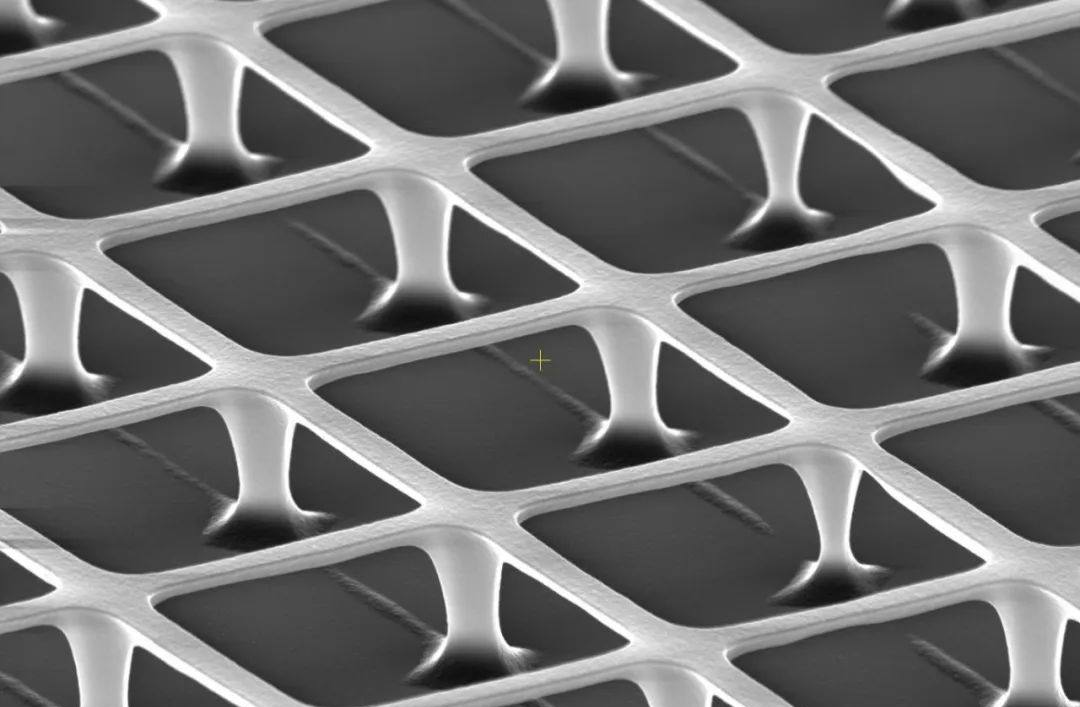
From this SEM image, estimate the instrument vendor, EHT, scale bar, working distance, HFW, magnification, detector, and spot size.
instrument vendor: Thermo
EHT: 30 kV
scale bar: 5000 nm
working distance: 16.8 mm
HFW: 25.9 µm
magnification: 16 K X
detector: ETD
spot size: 13